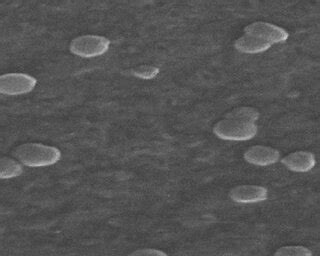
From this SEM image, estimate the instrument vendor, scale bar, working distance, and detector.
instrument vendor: FEI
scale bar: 200 nm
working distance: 9.81 mm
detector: SE